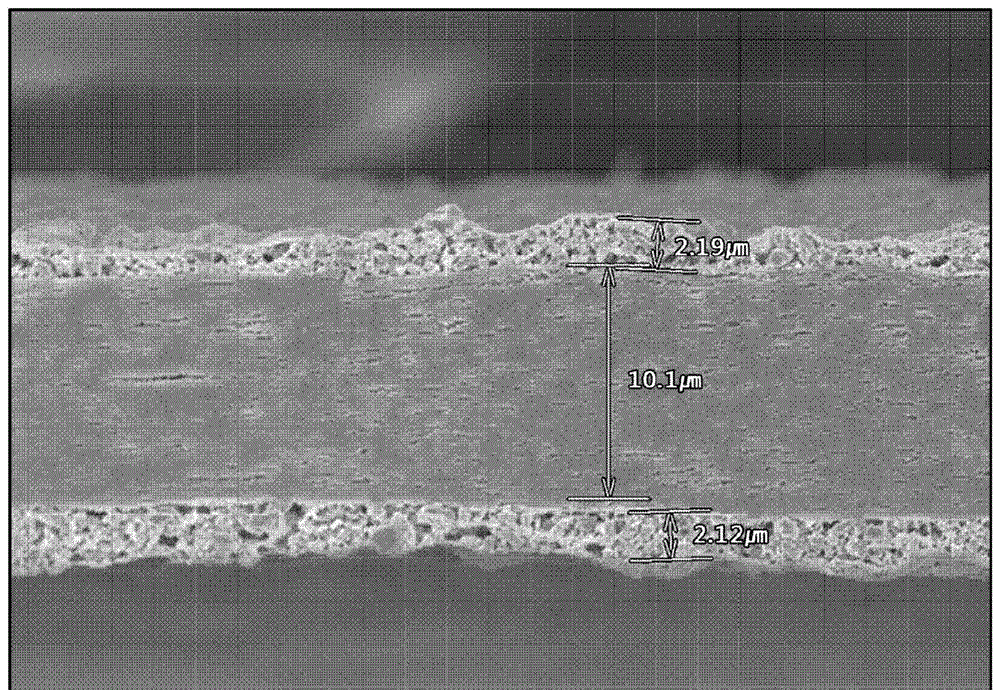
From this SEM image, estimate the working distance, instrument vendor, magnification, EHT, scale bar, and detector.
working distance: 8 mm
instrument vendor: Hitachi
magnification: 3 K X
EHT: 5 kV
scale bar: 10000 nm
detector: SE(U,LA0)